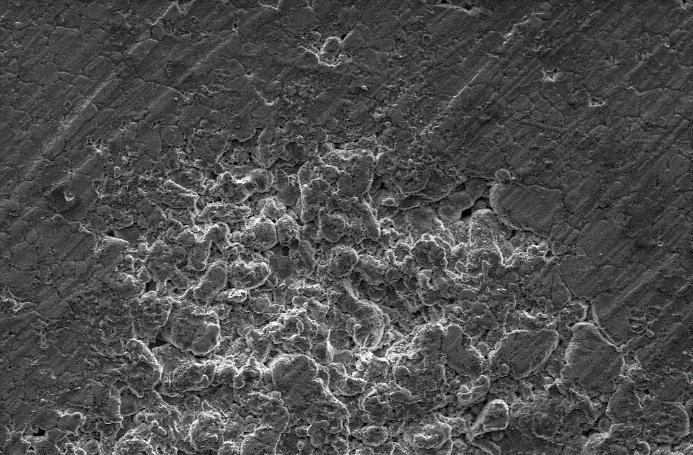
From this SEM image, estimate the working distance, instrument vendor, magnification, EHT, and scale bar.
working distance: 8.5 mm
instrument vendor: Zeiss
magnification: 0.548 K X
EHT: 10 kV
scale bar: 100000 nm